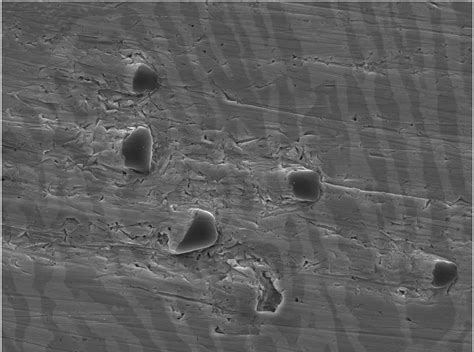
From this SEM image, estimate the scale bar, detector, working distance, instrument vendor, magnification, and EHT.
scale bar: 10000 nm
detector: SEI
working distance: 10.3 mm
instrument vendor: JEOL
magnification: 2.5 K X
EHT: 15 kV